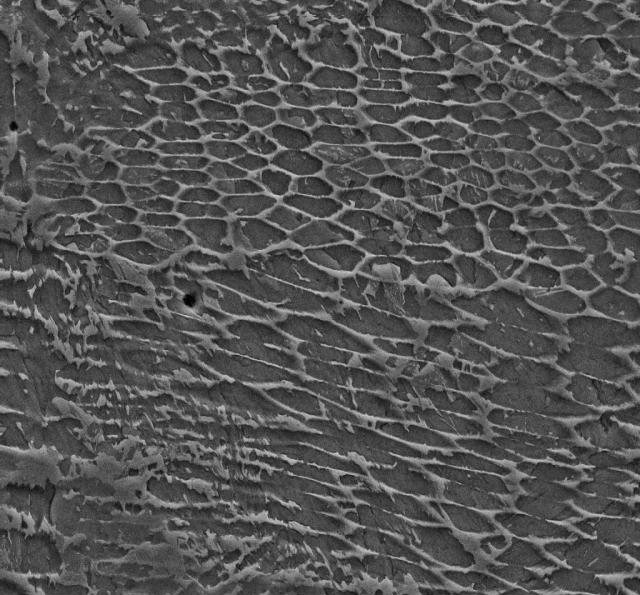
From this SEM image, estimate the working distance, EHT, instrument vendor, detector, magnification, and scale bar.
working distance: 8 mm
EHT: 15 kV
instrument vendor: Zeiss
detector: SE2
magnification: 10 K X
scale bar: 2000 nm